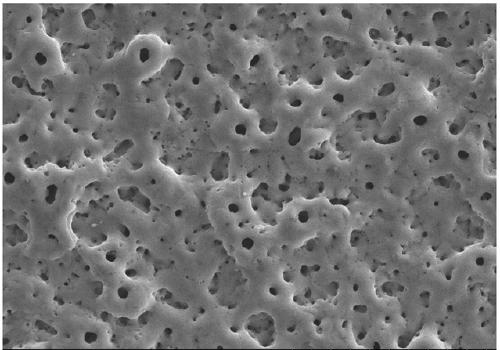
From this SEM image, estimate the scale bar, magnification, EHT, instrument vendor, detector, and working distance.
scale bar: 50000 nm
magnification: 1 K X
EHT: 20 kV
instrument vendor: Hitachi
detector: SE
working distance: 11.1 mm